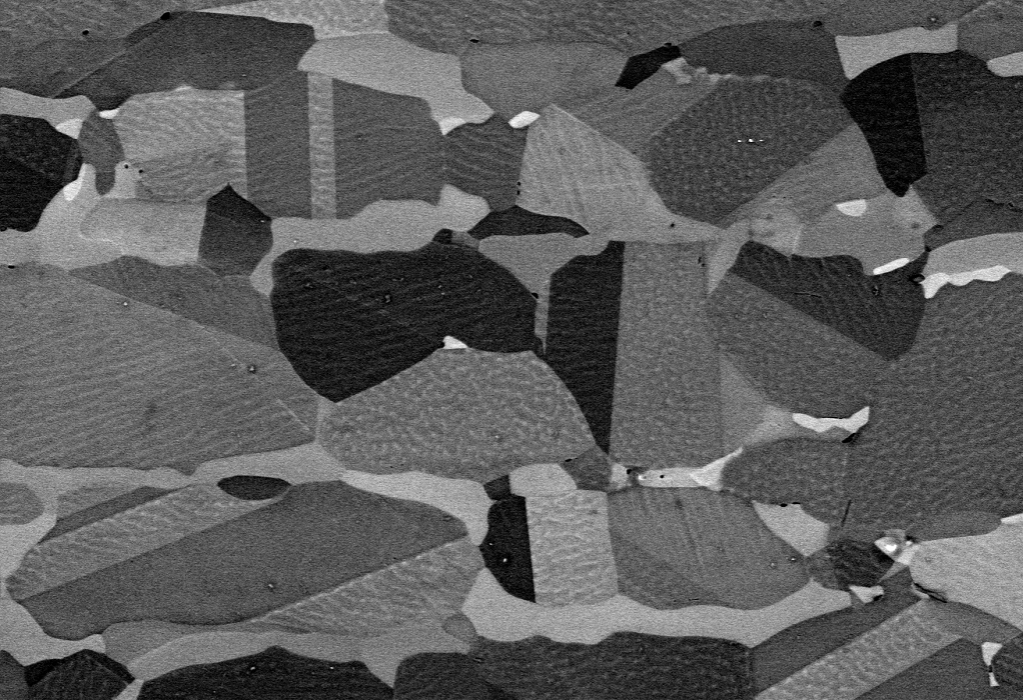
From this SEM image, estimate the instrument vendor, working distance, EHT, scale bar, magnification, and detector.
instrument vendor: Zeiss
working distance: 2.5 mm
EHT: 8 kV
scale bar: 2000 nm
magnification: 10 K X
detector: AsB